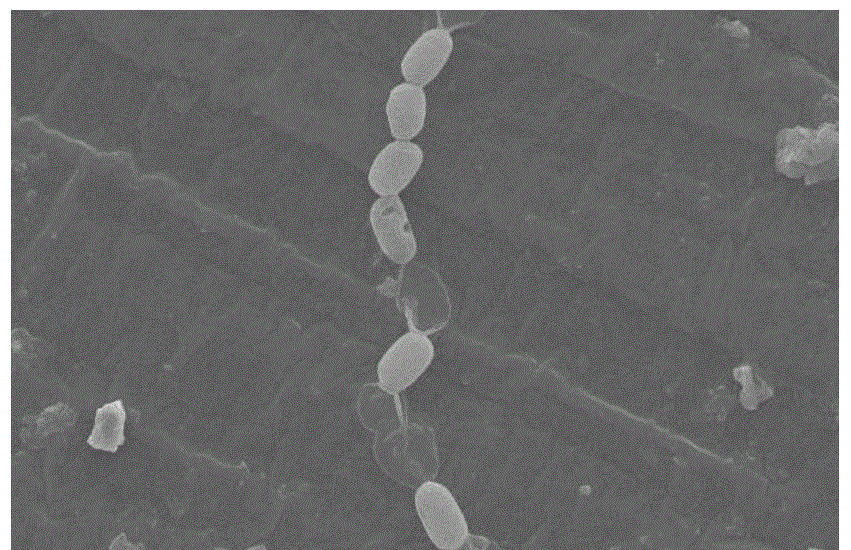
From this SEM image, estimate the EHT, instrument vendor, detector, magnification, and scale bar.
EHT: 10 kV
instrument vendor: JEOL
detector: SEI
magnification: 5 K X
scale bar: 5000 nm